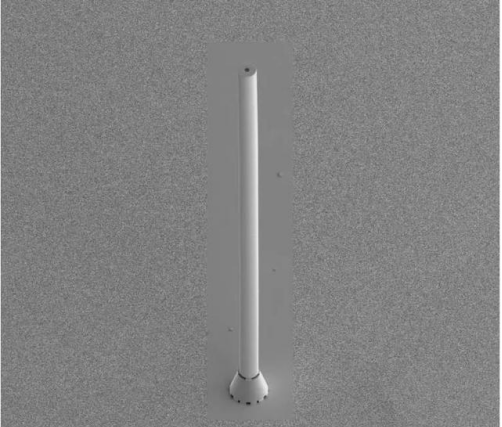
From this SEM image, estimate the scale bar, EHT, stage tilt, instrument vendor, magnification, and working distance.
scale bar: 500000 nm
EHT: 5 kV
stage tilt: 45°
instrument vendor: FEI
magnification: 0.12 K X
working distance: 9.7 mm